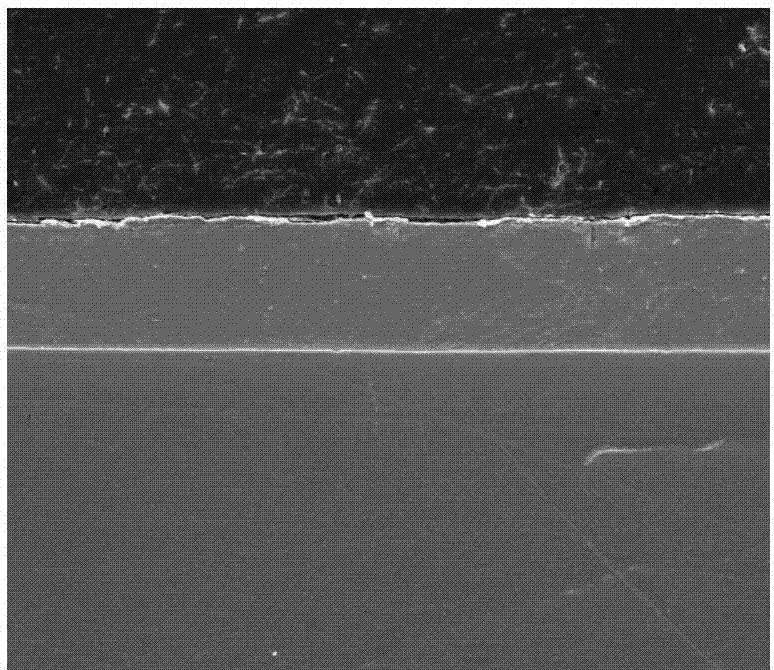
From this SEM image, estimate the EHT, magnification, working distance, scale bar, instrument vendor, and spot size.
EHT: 20 kV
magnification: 2.5 K X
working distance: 13.9 mm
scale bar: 50000 nm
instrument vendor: FEI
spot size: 4